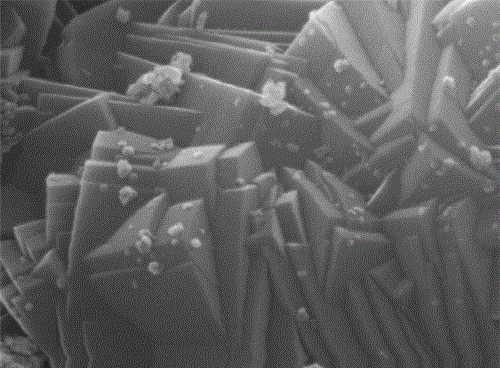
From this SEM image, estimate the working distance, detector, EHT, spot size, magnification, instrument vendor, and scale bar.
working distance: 10 mm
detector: ETD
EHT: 30 kV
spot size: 2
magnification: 65 K X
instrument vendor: FEI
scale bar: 1000 nm